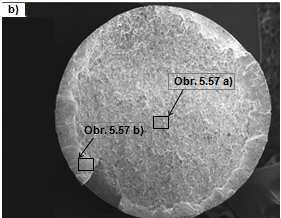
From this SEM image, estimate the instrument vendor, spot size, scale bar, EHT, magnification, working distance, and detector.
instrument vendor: FEI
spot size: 4.4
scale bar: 2000000 nm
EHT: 20 kV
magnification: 0.01 K X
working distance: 25 mm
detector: SE